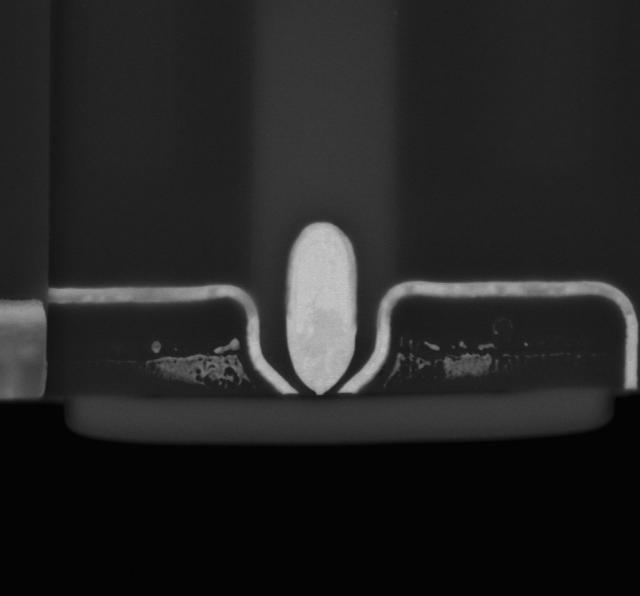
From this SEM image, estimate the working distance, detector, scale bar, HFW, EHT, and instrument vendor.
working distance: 2 mm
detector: MD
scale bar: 1000 nm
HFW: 3.63 µm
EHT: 7 kV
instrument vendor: FEI